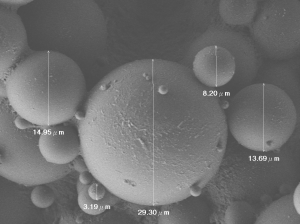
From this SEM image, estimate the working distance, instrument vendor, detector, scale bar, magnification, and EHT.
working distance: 7.8 mm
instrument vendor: JEOL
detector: LEI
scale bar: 10000 nm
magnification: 2 K X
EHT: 3 kV